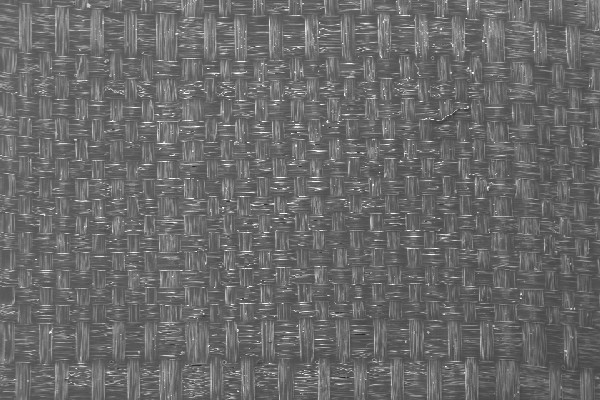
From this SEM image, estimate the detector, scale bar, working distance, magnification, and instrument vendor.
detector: SE1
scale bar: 200000 nm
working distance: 18.5 mm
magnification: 0.036 K X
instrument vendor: Zeiss